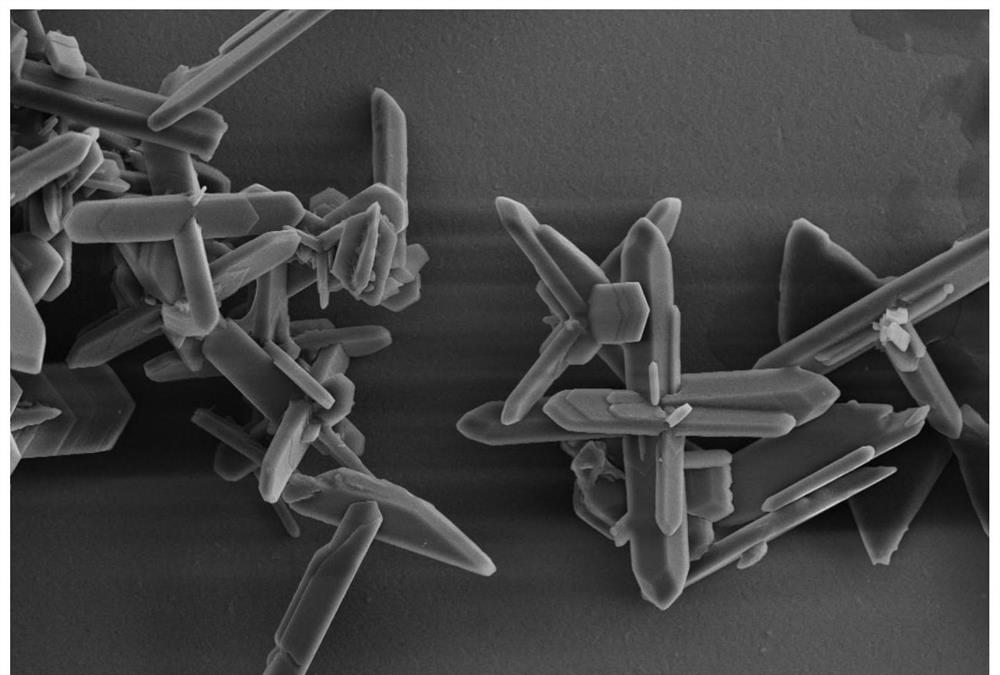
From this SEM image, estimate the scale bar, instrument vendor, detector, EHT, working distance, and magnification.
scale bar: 2000 nm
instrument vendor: Zeiss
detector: InLens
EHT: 5 kV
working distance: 4.5 mm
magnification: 3 K X